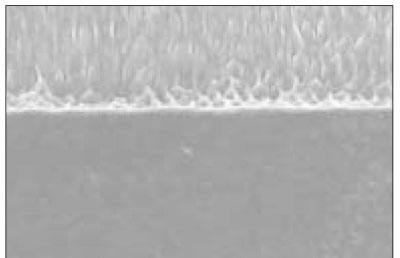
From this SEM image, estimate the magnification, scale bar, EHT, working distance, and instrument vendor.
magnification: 1 K X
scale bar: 50000 nm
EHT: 15 kV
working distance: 11.6 mm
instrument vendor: Hitachi